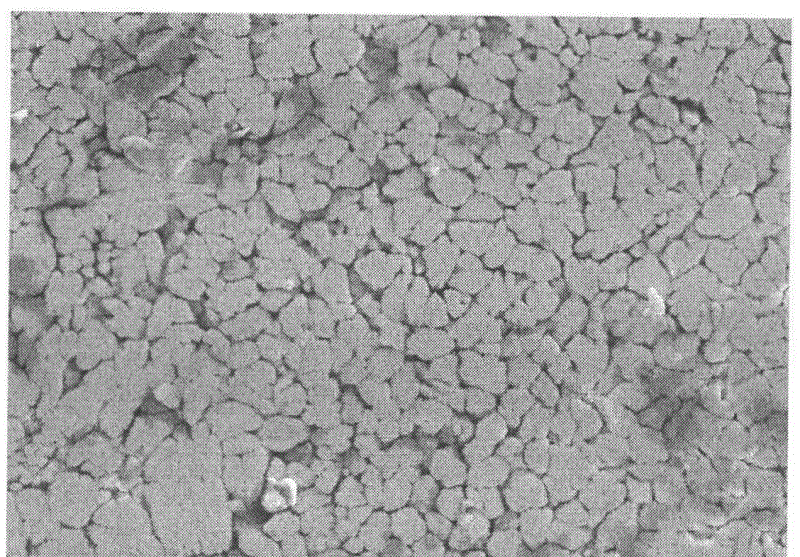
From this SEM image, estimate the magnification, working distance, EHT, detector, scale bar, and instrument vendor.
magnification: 5 K X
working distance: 10.7 mm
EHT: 10 kV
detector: SE(M)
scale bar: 10000 nm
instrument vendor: Hitachi